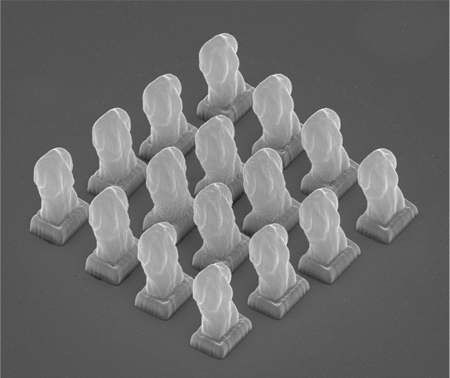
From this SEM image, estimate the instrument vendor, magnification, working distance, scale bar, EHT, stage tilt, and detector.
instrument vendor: FEI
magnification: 6.184 K X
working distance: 18.8 mm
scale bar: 20000 nm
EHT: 20 kV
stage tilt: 45°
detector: ETD